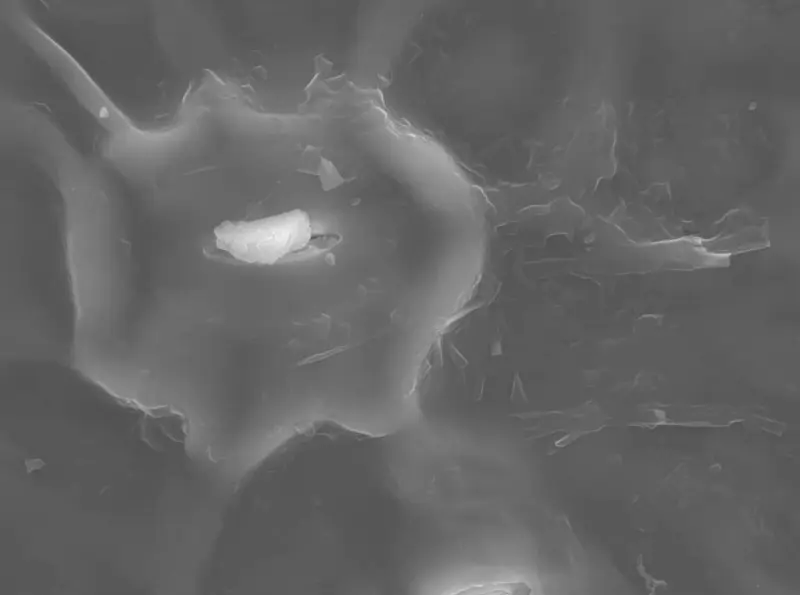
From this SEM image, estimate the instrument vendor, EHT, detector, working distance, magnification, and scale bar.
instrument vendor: JEOL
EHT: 20 kV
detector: SEI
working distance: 10 mm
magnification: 2 K X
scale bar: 10000 nm